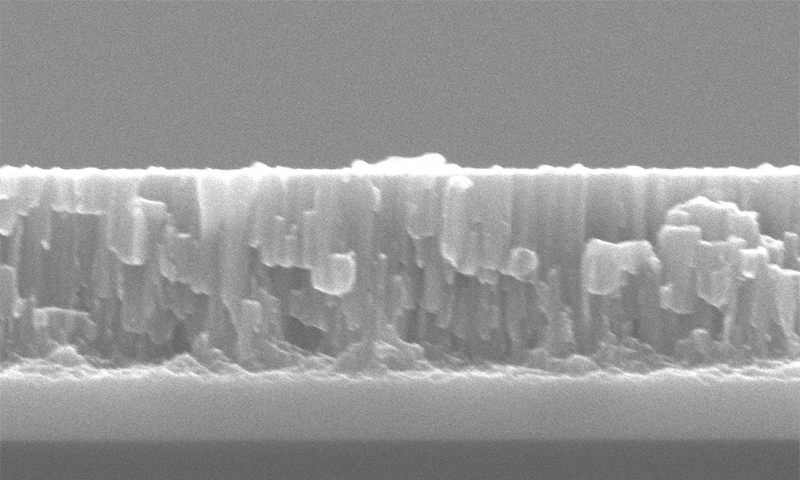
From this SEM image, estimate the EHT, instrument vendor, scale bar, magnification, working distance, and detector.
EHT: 10 kV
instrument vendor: JEOL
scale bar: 500 nm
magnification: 50 K X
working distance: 9.4 mm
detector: SED